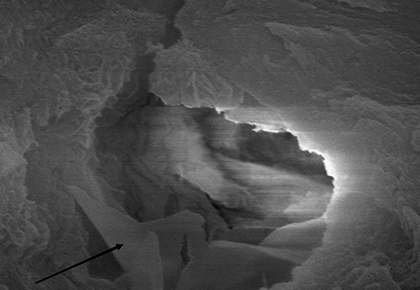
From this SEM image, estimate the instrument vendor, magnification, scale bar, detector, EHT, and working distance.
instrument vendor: Hitachi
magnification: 40 K X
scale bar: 1000 nm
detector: SE(M)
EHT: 15 kV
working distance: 15.1 mm